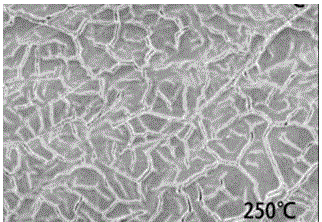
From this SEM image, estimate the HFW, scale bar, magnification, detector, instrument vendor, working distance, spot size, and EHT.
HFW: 49.7 µm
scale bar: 20000 nm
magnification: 6 K X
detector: ETD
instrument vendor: FEI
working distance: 11.2 mm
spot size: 2.5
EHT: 5 kV